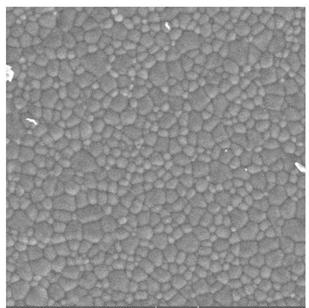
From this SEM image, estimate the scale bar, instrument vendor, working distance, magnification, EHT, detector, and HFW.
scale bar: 20000 nm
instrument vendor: Tescan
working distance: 10.1 mm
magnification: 2 K X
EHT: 30 kV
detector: SE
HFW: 142 µm